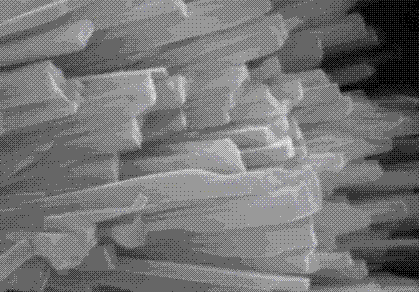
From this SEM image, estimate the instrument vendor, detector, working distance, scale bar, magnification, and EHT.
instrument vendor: Hitachi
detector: SE(M)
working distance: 16.9 mm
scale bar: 1000 nm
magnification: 40 K X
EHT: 15 kV